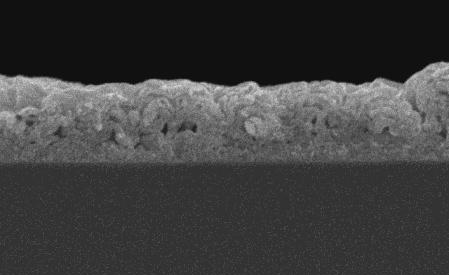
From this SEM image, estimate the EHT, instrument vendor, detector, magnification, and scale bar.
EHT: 20 kV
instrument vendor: JEOL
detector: SEI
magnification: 5 K X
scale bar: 5000 nm